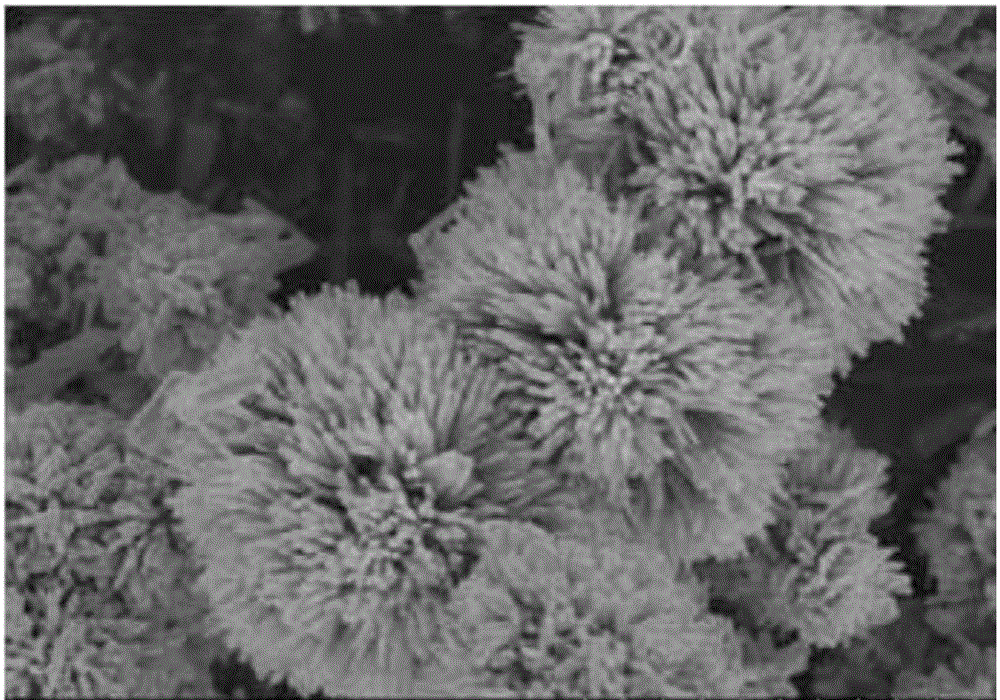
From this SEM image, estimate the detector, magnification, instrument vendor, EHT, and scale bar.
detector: SE(M)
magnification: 10 K X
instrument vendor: Hitachi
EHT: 10 kV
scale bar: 5000 nm